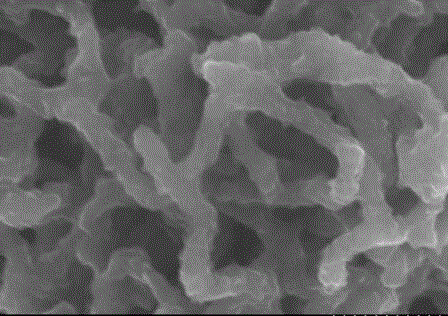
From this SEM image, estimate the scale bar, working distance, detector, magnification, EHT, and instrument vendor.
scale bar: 500 nm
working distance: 9.4 mm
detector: SE(M)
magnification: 60 K X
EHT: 5 kV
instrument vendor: Hitachi